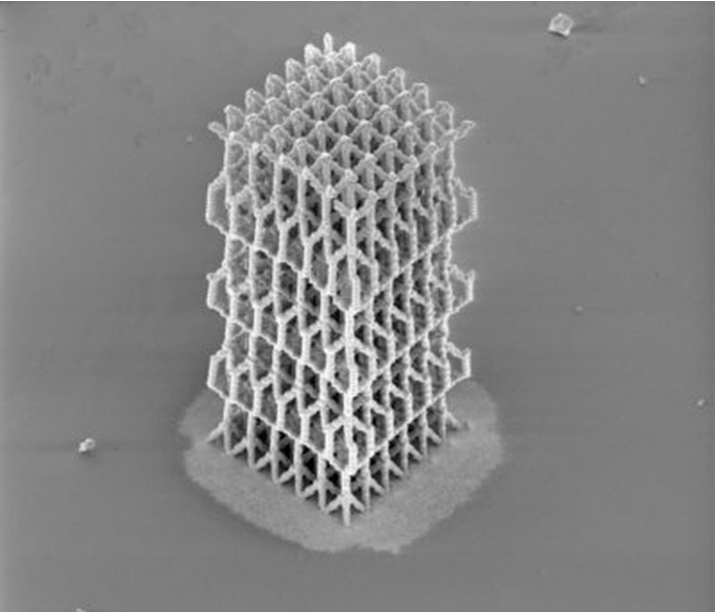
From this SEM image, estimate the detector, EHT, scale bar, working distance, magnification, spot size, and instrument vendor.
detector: ETD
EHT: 2 kV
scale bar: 2000000 nm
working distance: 29.4 mm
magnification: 0.025 K X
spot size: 3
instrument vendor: FEI